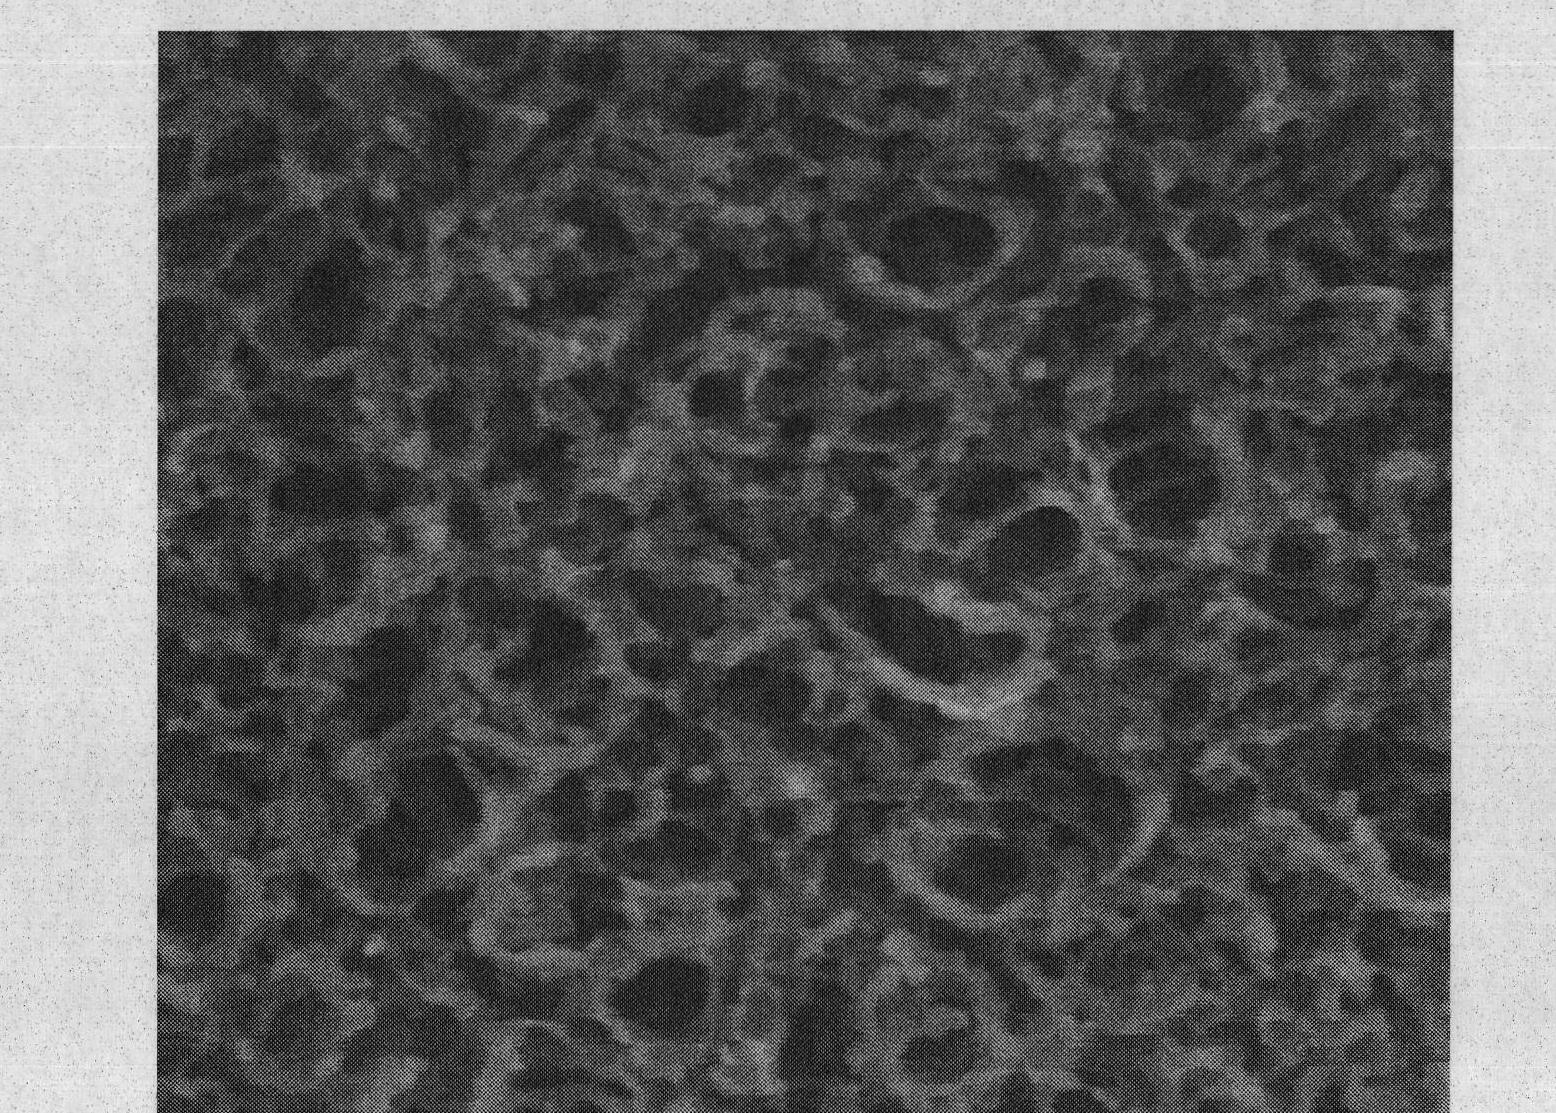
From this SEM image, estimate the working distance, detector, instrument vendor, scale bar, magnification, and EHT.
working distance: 6.1 mm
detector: TLD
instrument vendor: FEI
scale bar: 300 nm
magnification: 200 K X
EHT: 10 kV